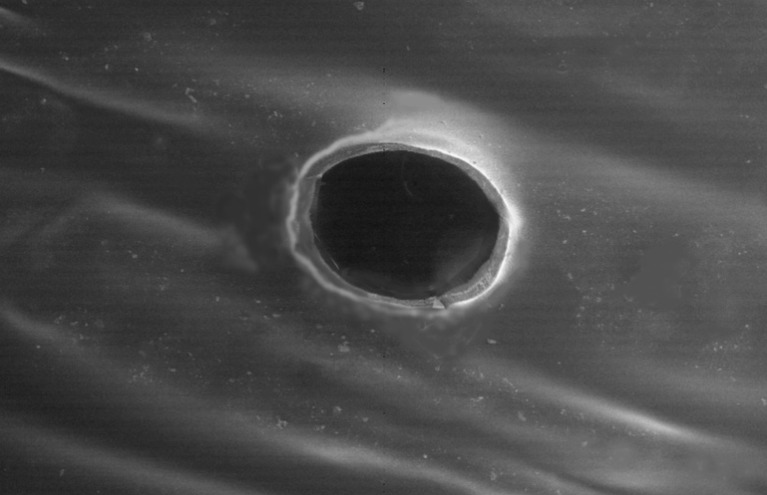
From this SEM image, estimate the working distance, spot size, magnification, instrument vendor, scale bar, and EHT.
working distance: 9.3 mm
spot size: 3.5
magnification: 0.1 K X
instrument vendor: FEI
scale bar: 1000000 nm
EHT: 5 kV